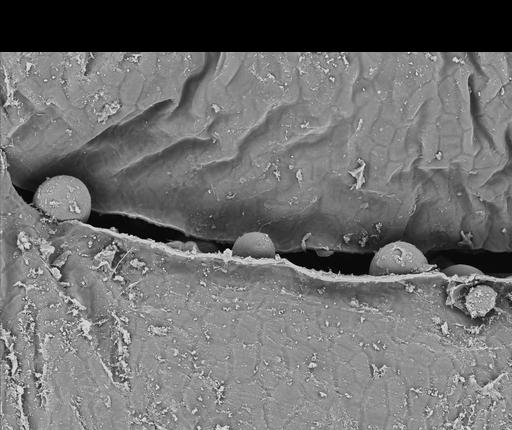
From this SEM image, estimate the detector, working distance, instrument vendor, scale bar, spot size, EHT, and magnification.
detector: BSE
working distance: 8.4 mm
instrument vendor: FEI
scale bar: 100000 nm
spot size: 3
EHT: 15 kV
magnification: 0.245 K X